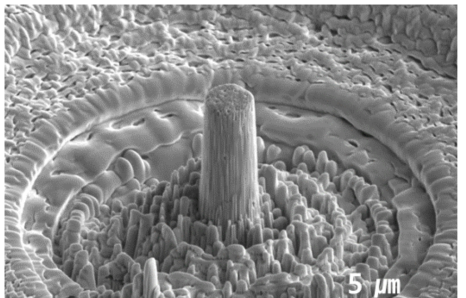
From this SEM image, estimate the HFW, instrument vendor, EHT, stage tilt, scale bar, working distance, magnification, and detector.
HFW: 20.7 µm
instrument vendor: FEI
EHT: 5 kV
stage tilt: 52°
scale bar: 5000 nm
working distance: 4 mm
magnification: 10 K X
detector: ETD